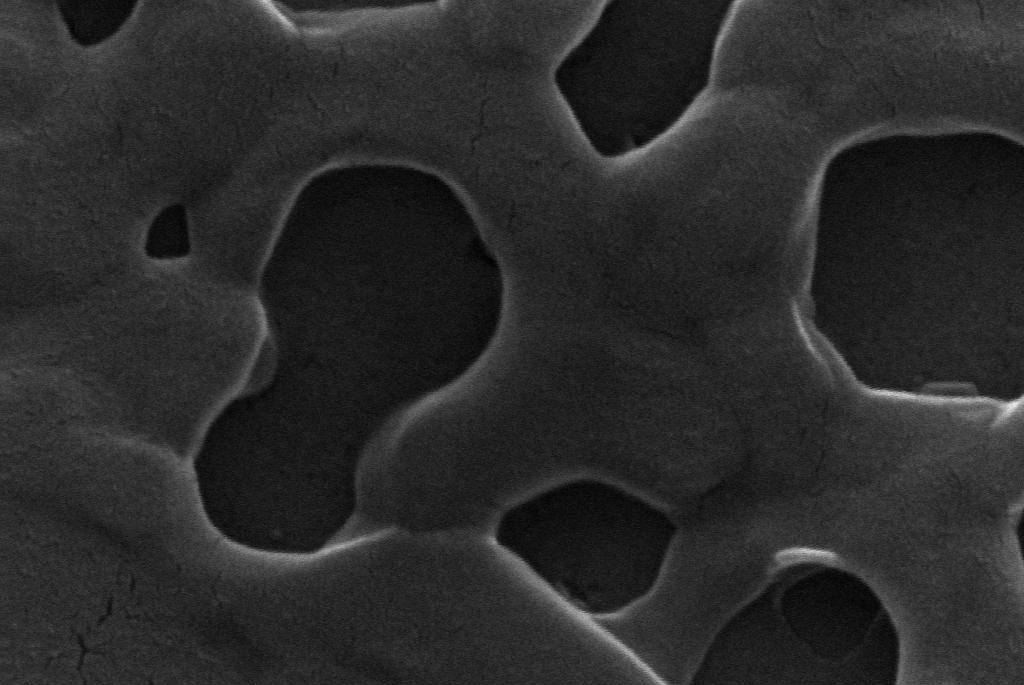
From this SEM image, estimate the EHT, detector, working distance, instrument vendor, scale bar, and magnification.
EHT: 20 kV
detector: SE1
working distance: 10 mm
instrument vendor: Zeiss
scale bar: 200 nm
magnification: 30 K X